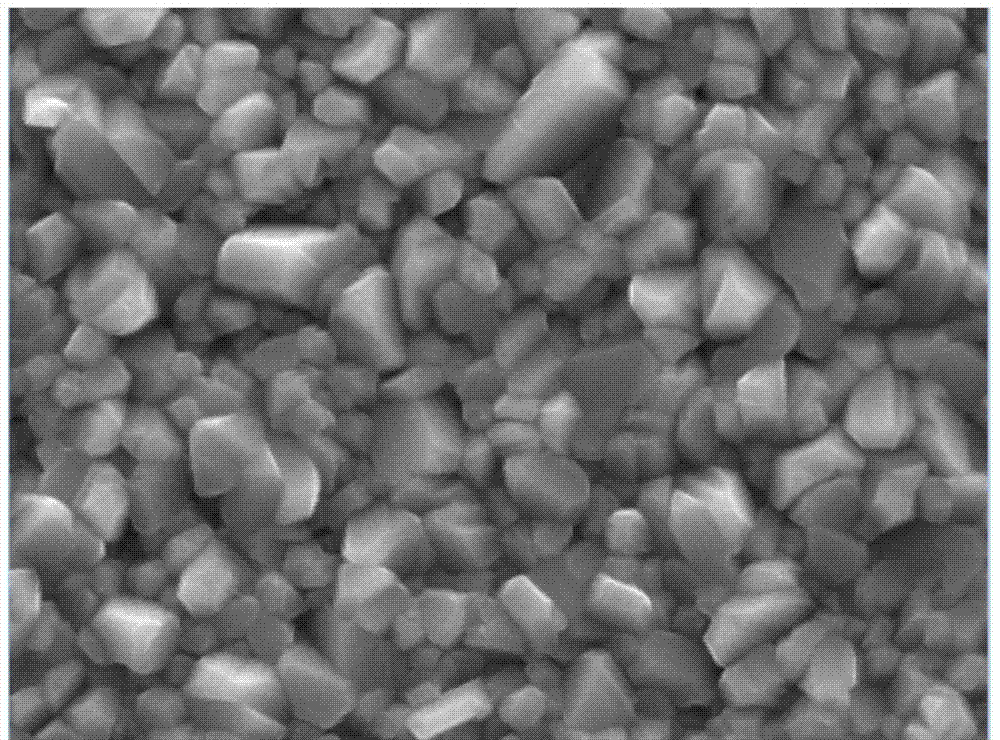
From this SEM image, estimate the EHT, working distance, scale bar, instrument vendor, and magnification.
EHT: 20 kV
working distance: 14.87 mm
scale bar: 2000 nm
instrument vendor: Tescan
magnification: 20 K X